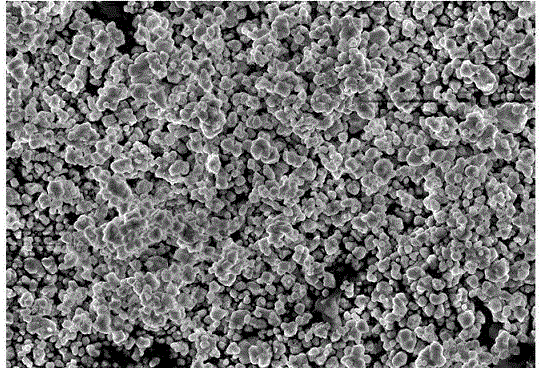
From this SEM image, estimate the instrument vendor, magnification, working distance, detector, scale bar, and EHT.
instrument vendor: Zeiss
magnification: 20 K X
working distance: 6.6 mm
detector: InLens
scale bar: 1000 nm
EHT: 5 kV